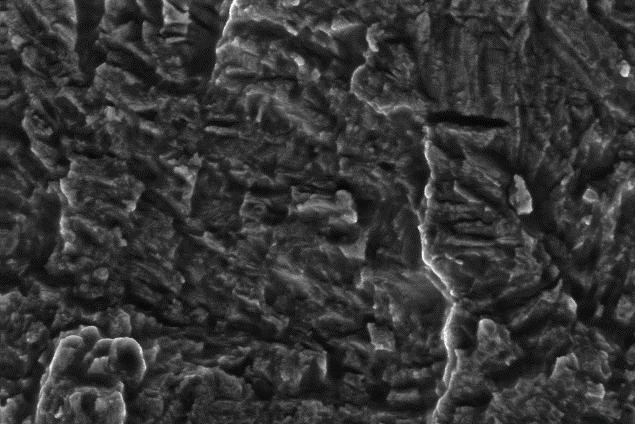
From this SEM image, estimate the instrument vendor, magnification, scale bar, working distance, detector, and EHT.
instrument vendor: Zeiss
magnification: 2.5 K X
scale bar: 10000 nm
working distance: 23 mm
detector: SE1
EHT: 20 kV